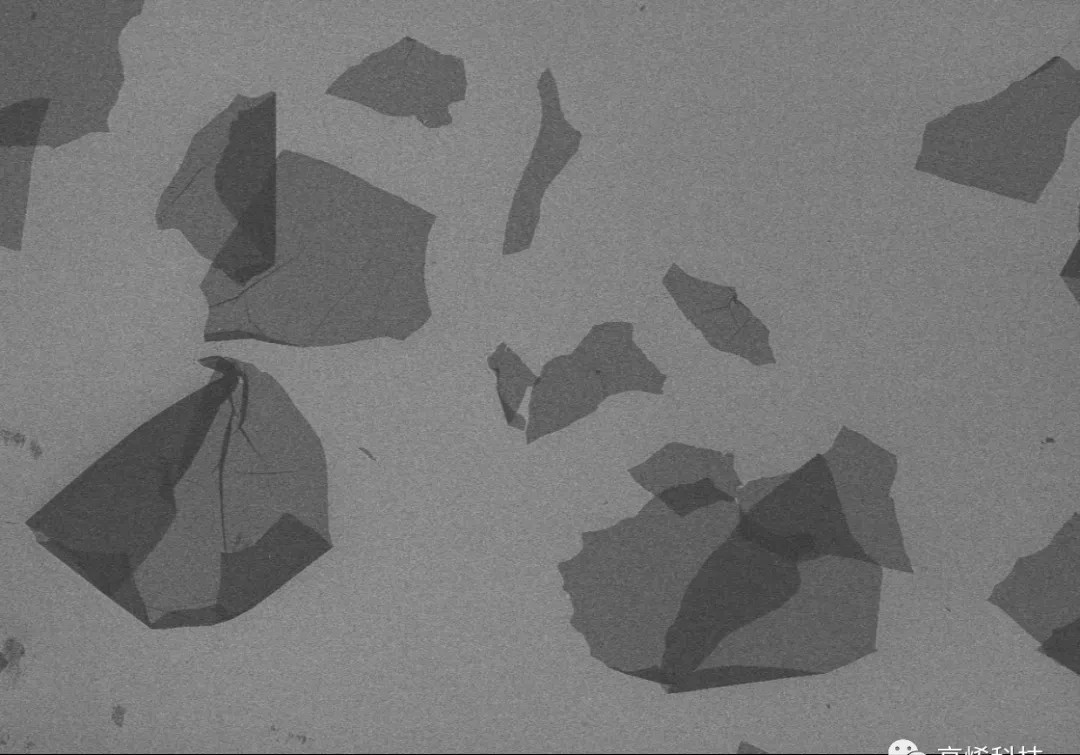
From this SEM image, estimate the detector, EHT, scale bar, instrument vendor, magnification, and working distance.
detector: SE(M)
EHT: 3 kV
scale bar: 100000 nm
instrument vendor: Hitachi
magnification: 0.3 K X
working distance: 10.8 mm